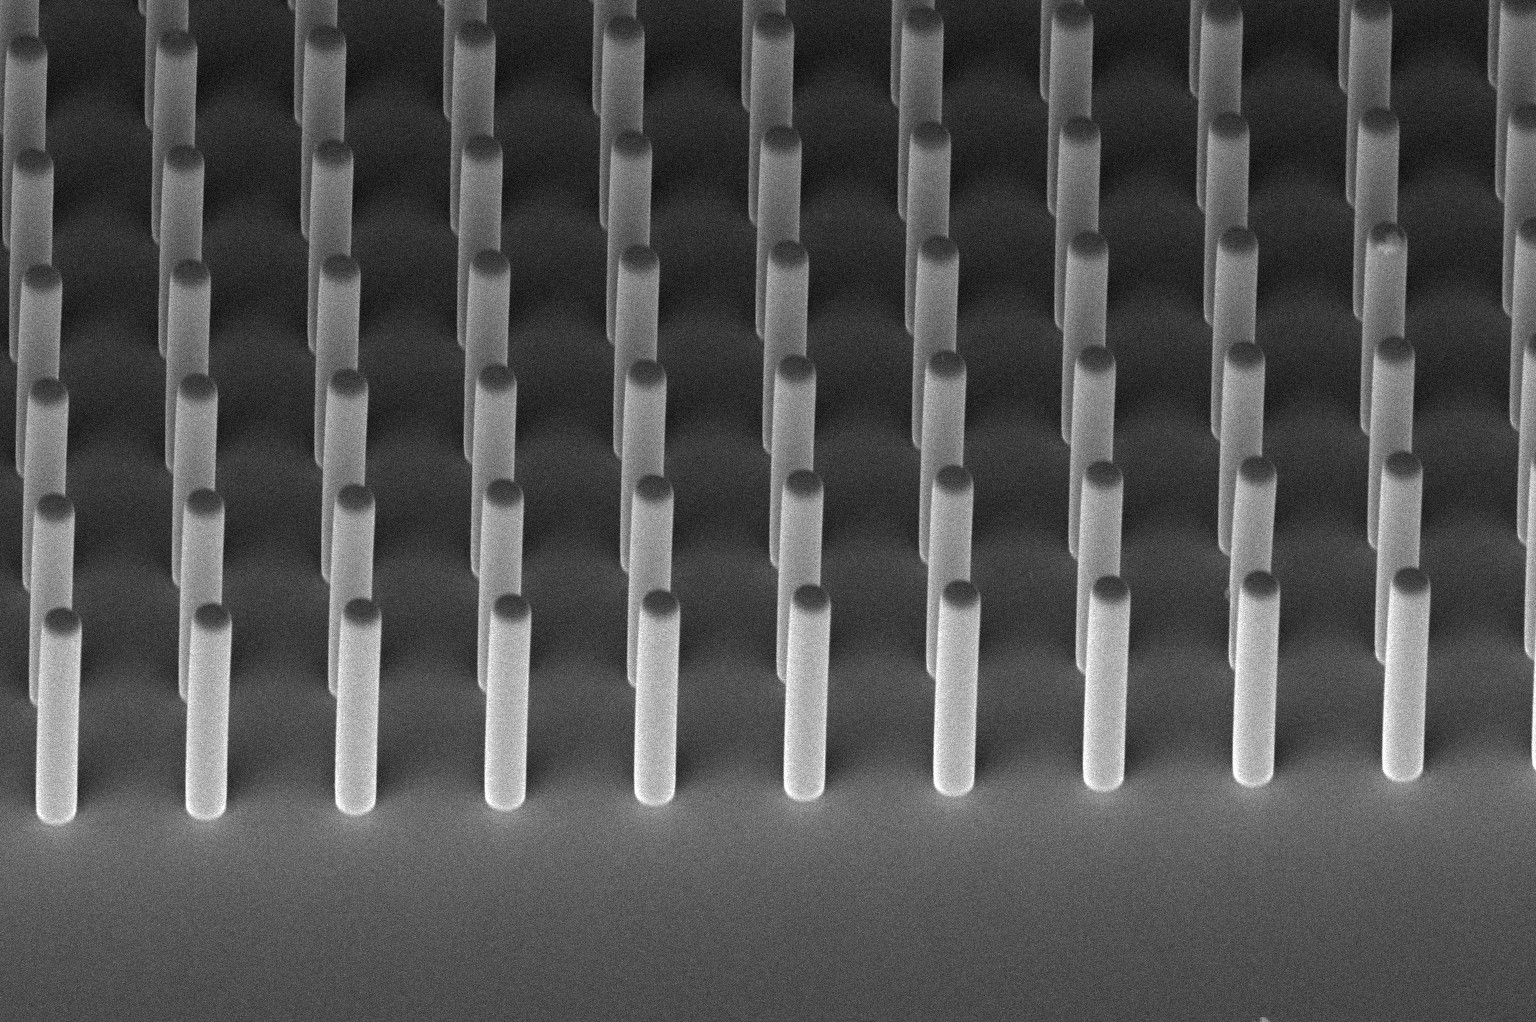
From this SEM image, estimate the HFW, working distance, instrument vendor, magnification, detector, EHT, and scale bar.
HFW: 259 µm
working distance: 3.9 mm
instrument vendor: FEI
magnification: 0.8 K X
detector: TLD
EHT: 2 kV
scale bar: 50000 nm